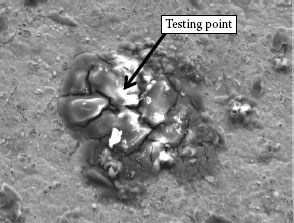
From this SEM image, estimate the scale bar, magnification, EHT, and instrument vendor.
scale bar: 10000 nm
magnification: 1.2 K X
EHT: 15 kV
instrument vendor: other